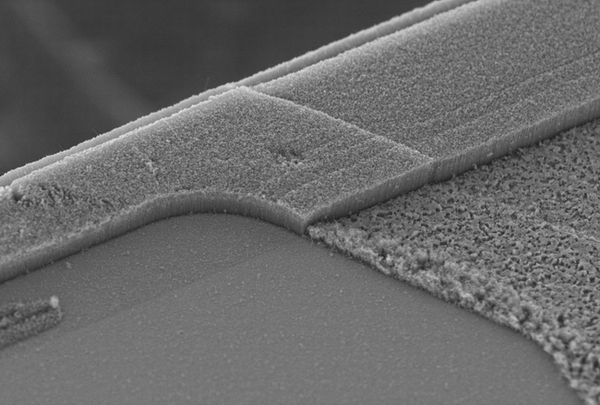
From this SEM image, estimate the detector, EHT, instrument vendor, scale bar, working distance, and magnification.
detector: SE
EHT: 5 kV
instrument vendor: Hitachi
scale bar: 50000 nm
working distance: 11.4 mm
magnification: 1 K X